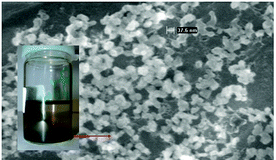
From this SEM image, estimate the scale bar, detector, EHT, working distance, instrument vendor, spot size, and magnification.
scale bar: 200 nm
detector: TLD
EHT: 5 kV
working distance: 4.6 mm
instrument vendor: FEI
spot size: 4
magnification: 200 K X